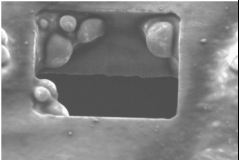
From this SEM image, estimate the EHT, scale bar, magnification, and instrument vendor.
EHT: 10 kV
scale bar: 10000 nm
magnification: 1.3 K X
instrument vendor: JEOL